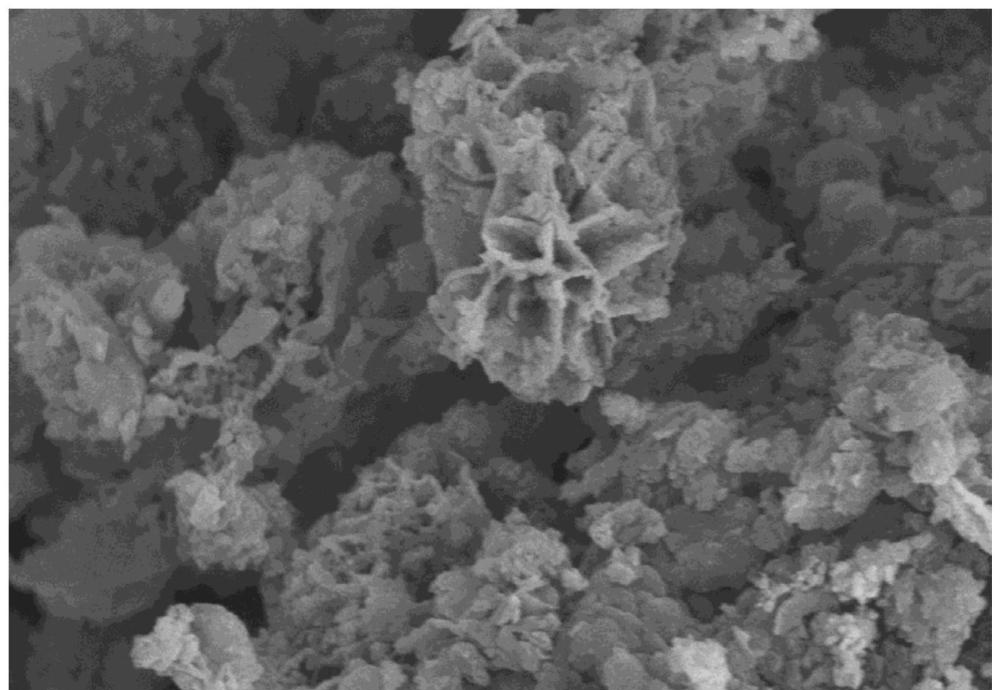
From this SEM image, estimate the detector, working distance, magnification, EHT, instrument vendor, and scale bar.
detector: SE(M)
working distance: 9.7 mm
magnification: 20 K X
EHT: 5 kV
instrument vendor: Hitachi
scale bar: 2000 nm